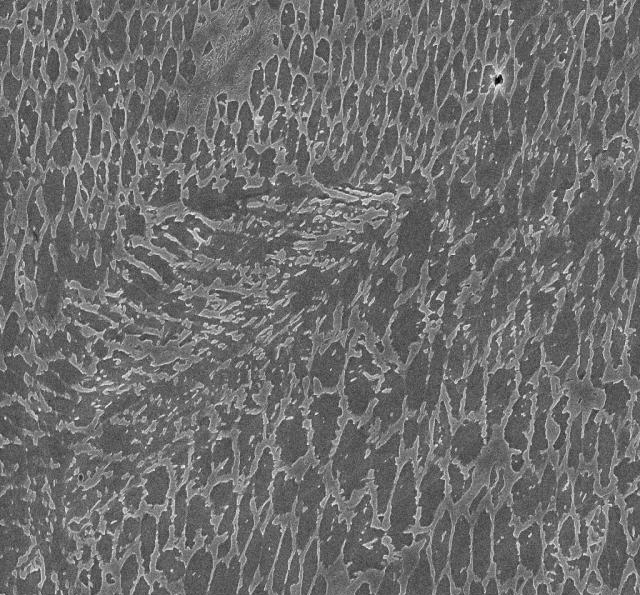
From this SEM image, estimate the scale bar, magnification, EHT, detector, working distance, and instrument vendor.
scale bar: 2000 nm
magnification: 10 K X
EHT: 15 kV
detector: InLens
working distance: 8 mm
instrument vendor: Zeiss